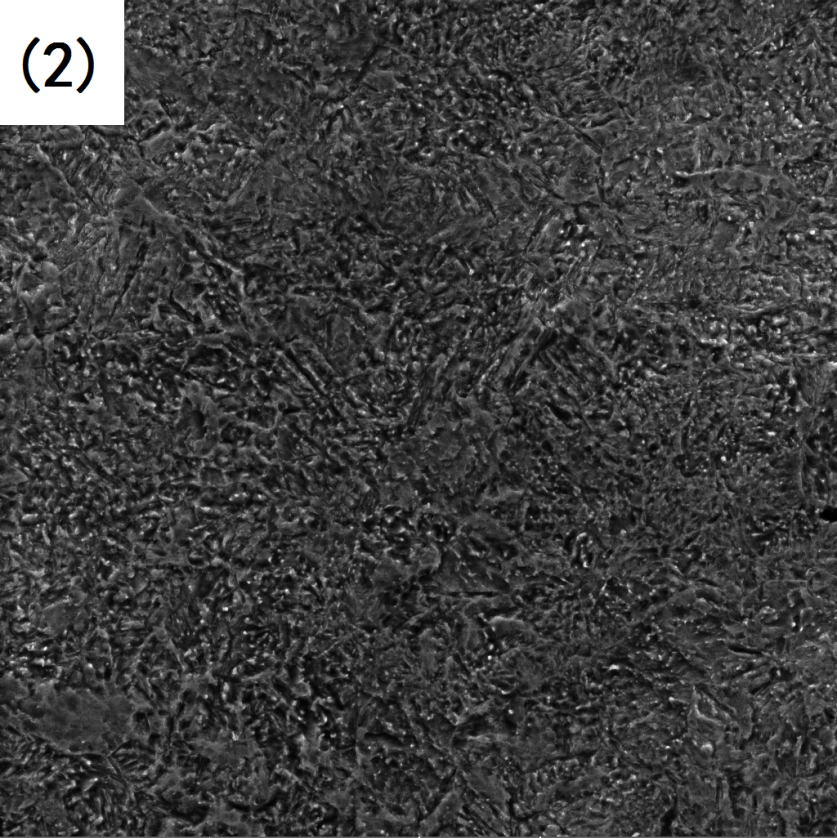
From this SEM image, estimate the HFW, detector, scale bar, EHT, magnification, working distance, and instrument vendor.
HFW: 138 µm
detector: SE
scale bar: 20000 nm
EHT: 25 kV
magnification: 2 K X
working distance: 22.42 mm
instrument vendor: Tescan